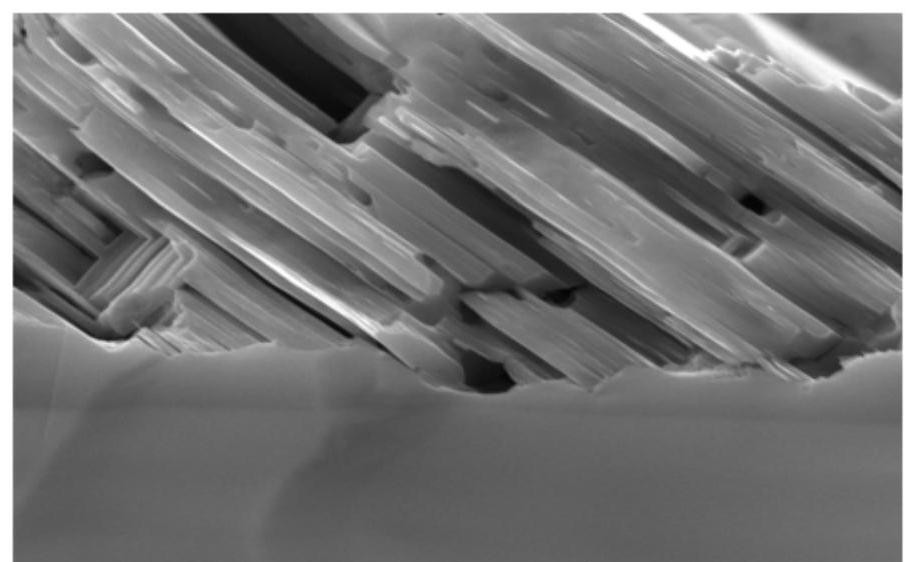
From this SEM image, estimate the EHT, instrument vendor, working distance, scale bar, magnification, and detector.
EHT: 20 kV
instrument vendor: Tescan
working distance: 14.52 mm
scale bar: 2000 nm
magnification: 20 K X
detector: SE